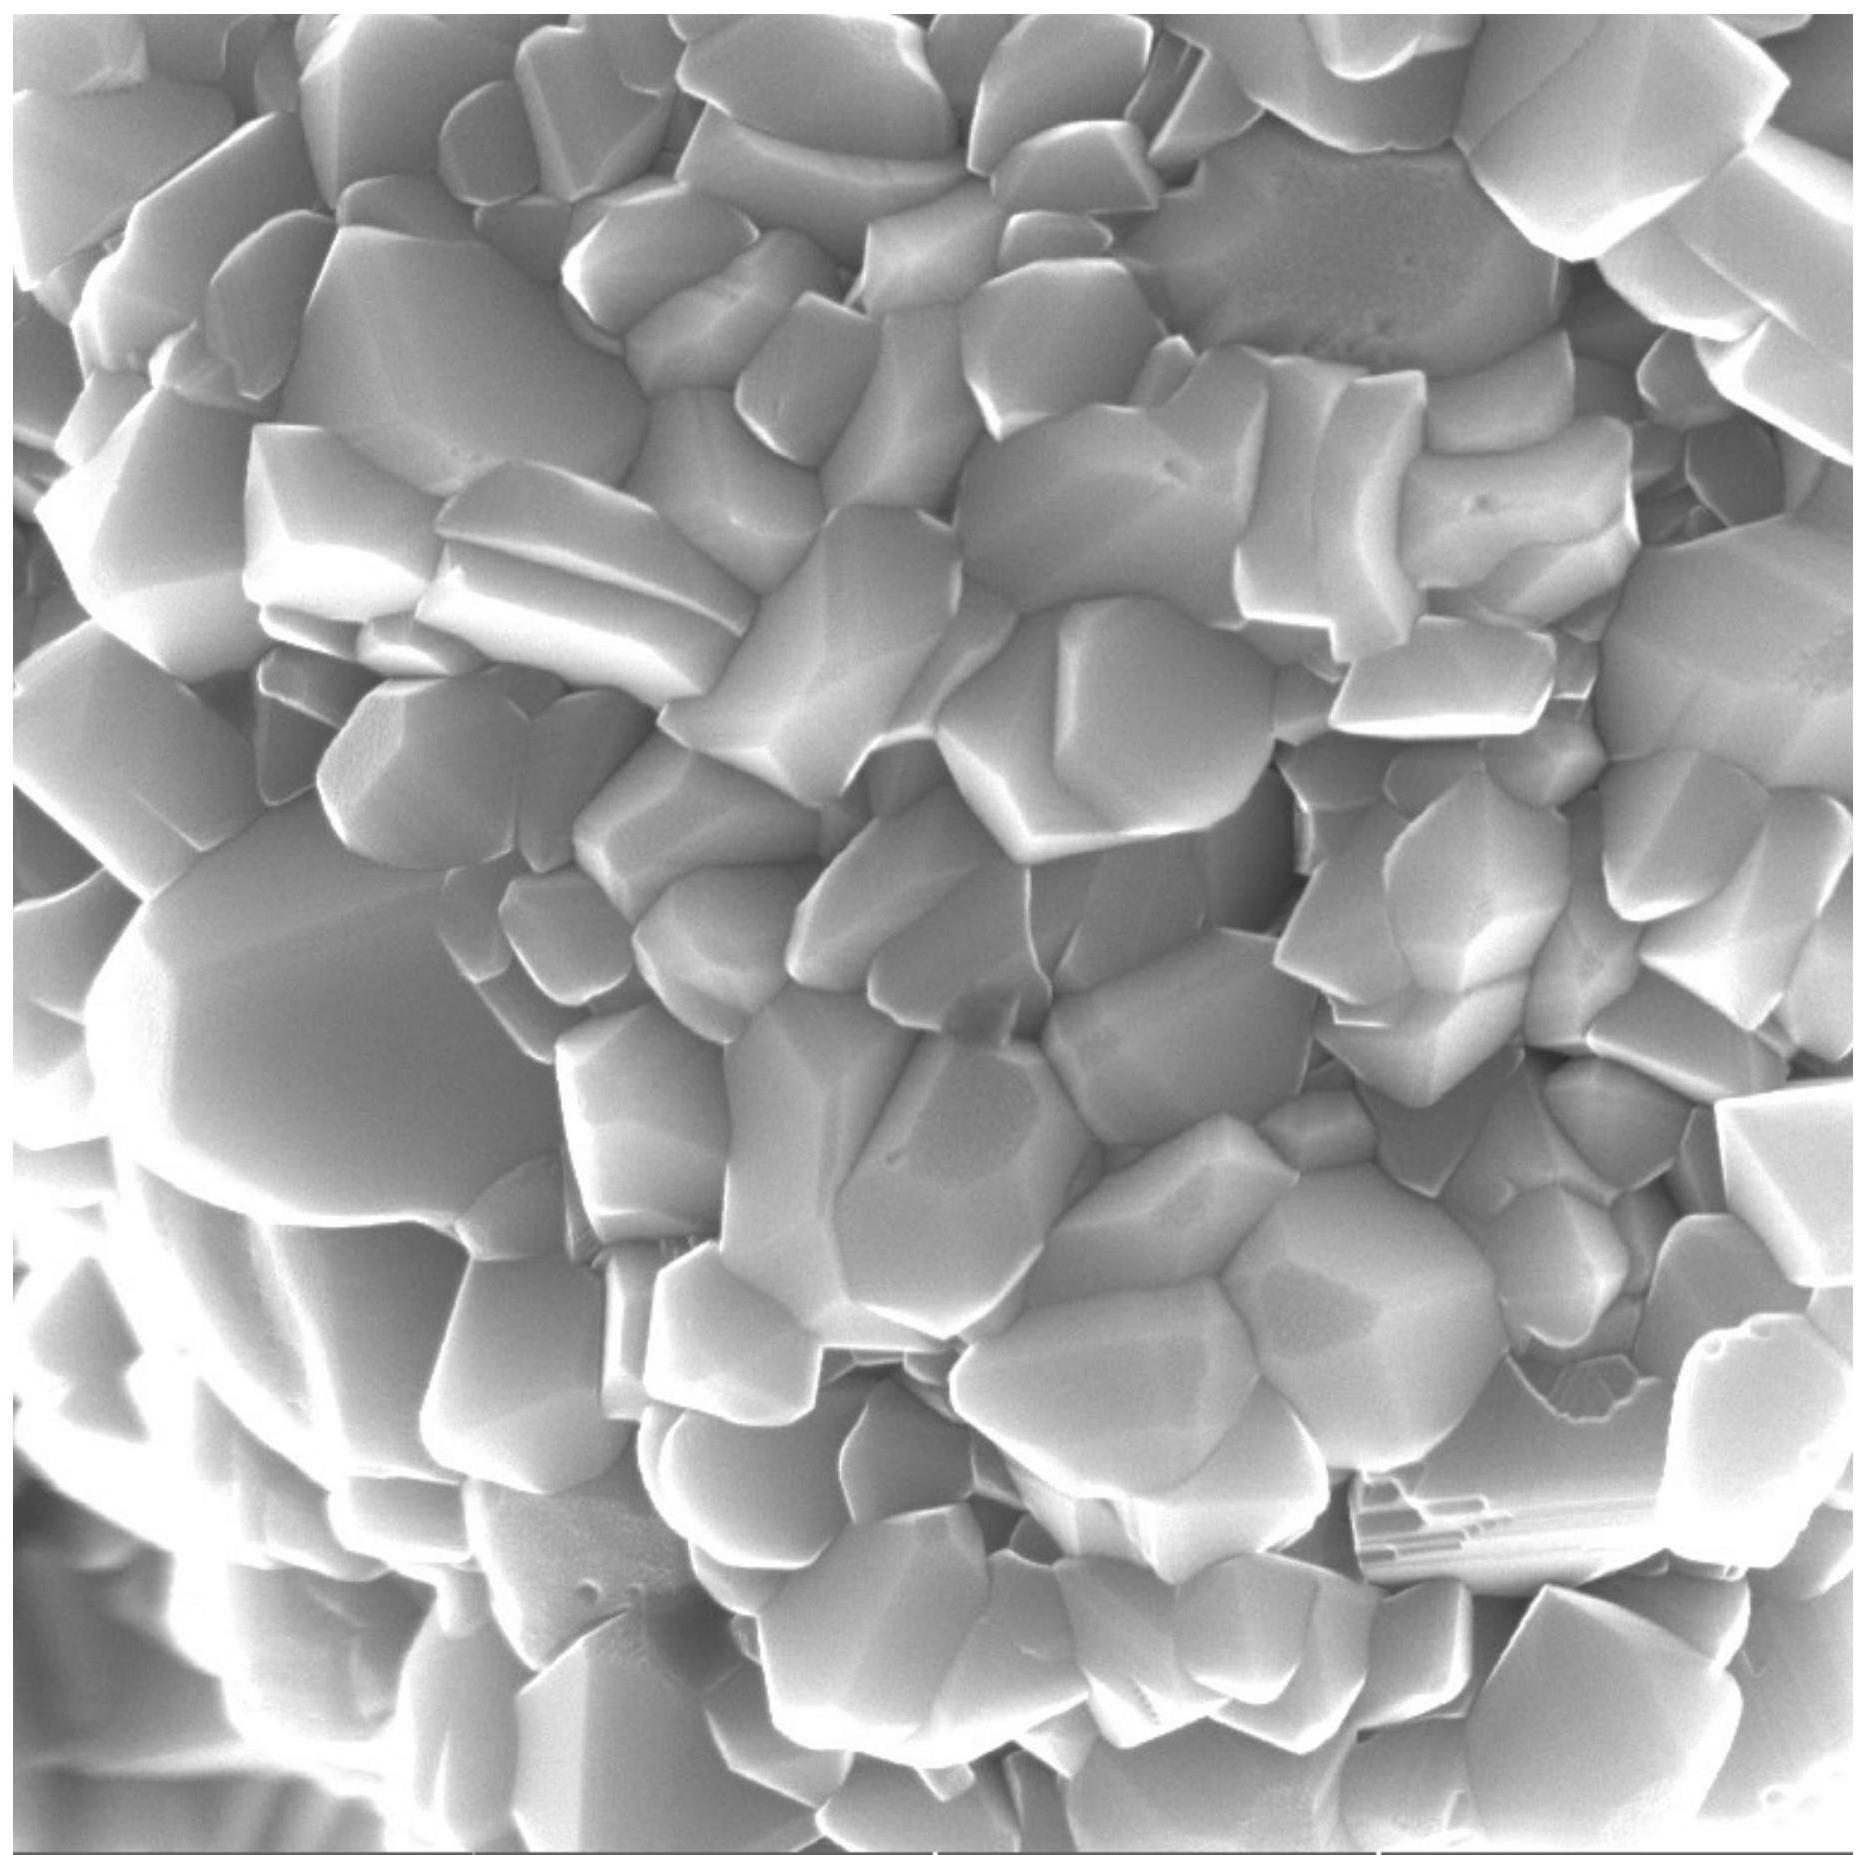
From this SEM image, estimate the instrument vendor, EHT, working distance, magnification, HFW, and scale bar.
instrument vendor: Tescan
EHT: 10 kV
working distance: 6.92 mm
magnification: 50 K X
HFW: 4.15 µm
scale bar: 1000 nm